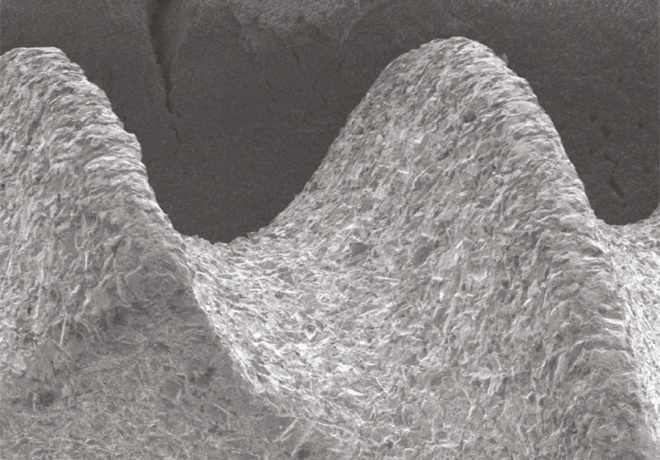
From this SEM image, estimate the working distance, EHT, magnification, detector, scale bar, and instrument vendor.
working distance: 15.4 mm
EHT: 5 kV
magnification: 0.08 K X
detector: SE(L)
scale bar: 500000 nm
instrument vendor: Hitachi